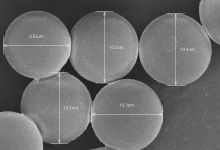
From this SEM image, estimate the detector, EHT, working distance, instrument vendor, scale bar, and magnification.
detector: SE(M)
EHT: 3 kV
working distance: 9.8 mm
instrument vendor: Hitachi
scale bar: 10000 nm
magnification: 4 K X